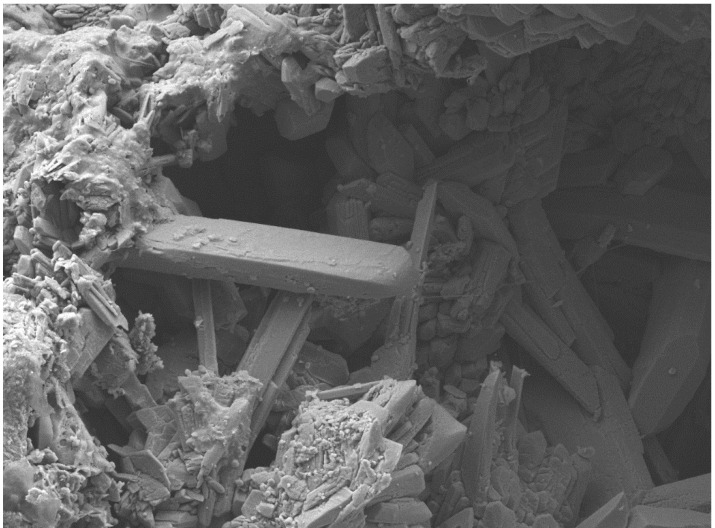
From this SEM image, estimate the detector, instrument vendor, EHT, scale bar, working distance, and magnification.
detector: SEI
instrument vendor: JEOL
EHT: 15 kV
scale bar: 100000 nm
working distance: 15 mm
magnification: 0.25 K X